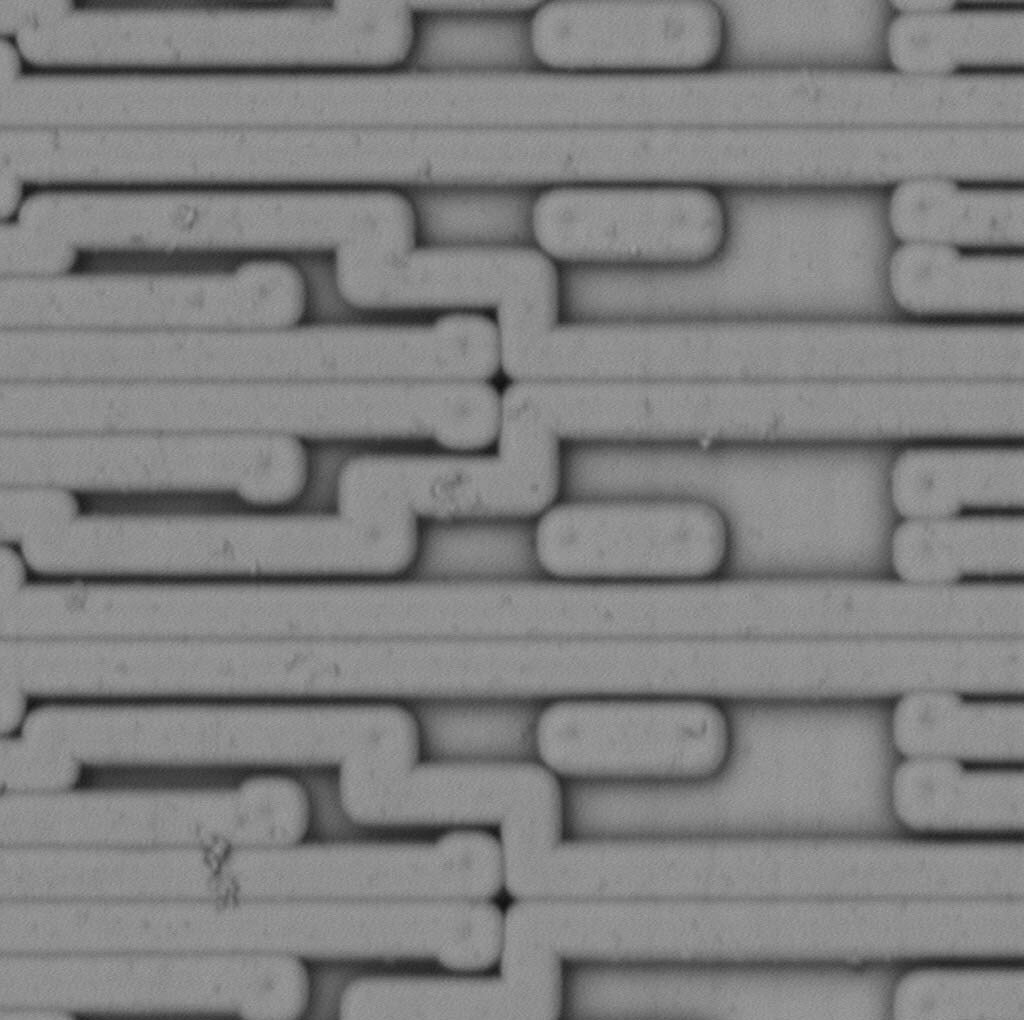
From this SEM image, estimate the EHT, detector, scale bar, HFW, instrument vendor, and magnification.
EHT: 5 kV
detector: BSD Full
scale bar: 8000 nm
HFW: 31.2 µm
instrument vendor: other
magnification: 8.6 K X